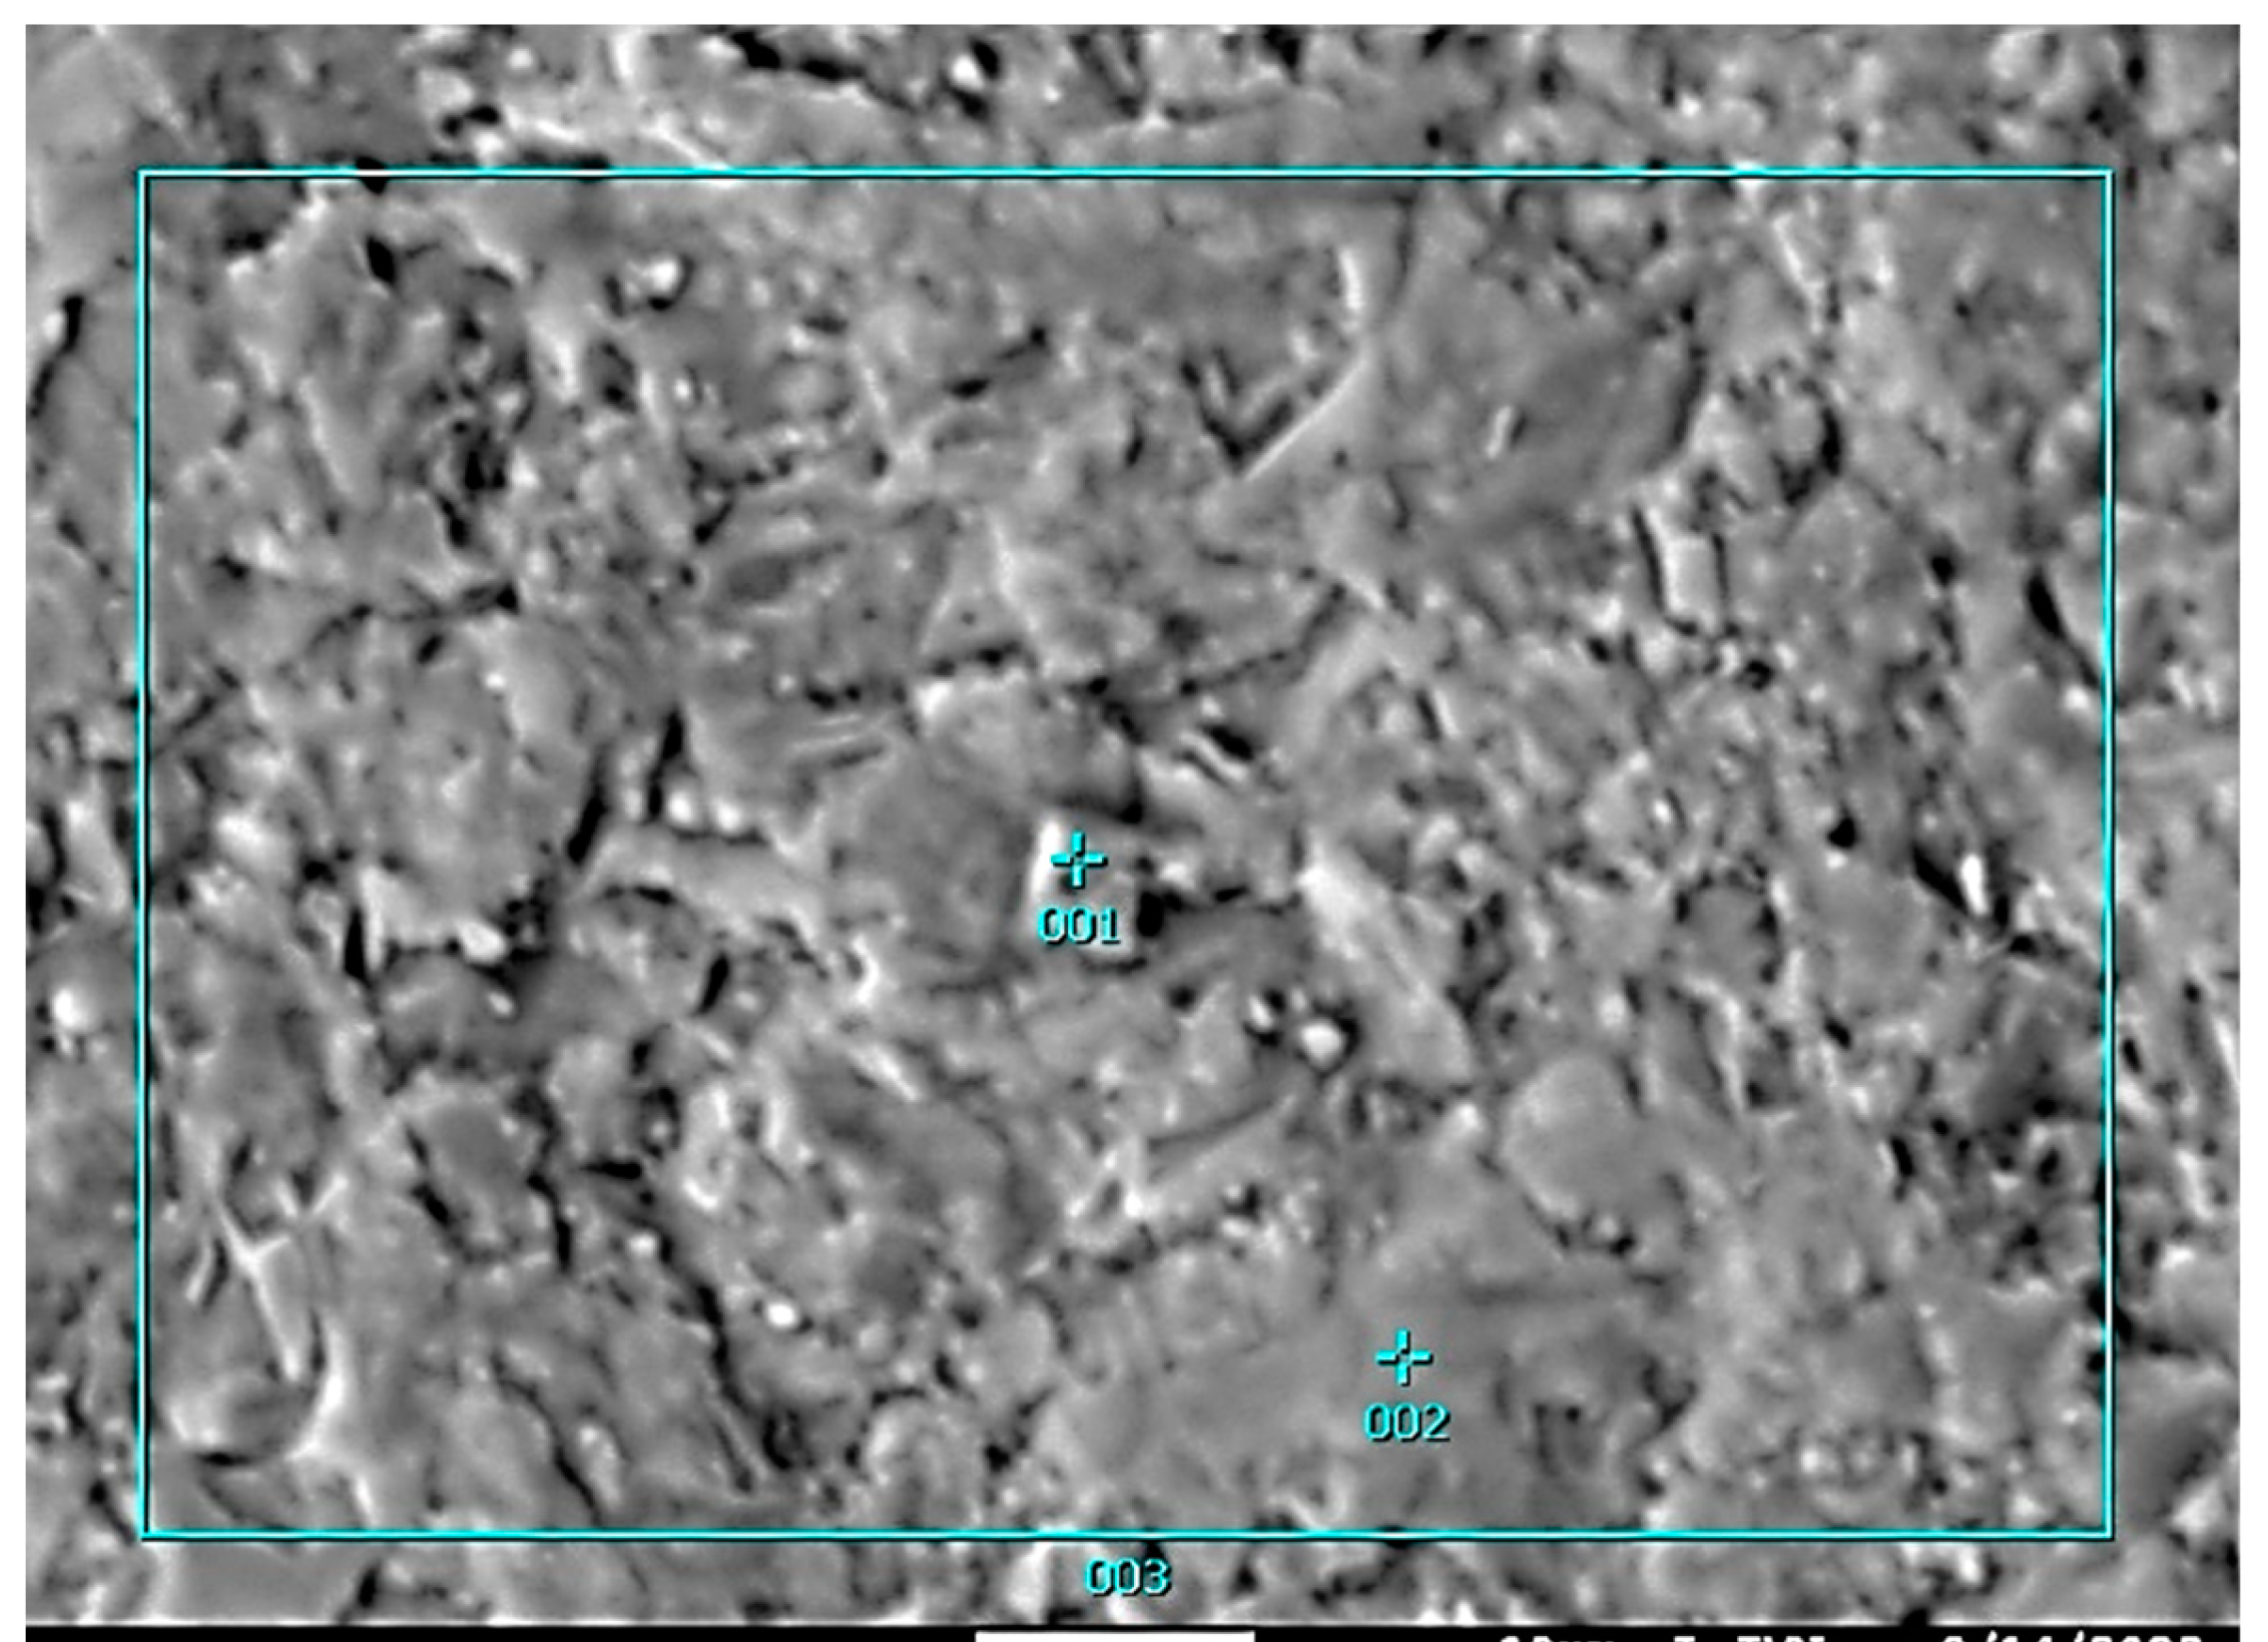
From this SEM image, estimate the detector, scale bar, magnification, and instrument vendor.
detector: COMPO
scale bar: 10000 nm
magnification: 1.5 K X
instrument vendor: JEOL